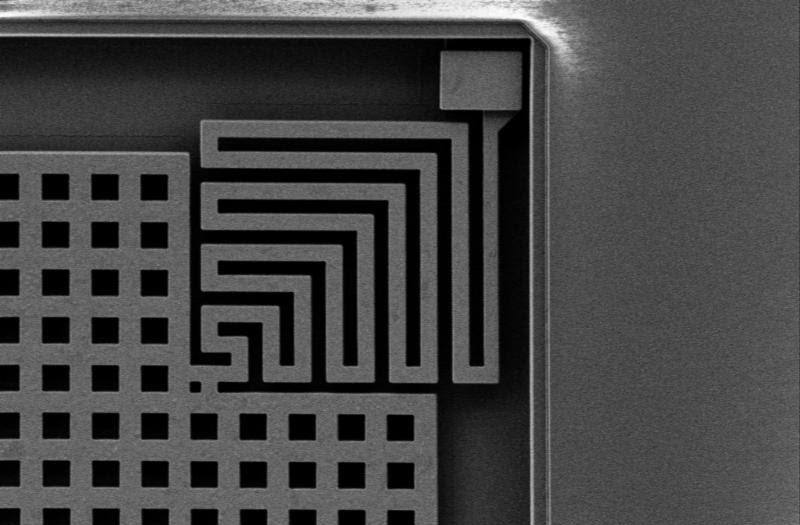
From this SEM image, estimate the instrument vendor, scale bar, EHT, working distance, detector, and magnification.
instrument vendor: Zeiss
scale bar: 10000 nm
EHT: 3 kV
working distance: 5.7 mm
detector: SE2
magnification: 4 K X